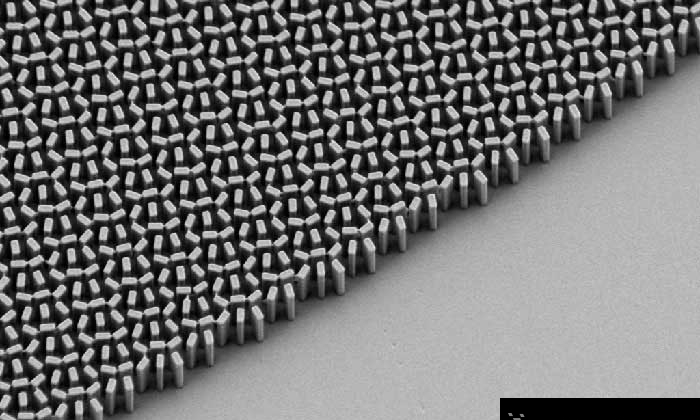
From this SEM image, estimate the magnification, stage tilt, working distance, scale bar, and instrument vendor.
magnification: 22.81 K X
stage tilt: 35°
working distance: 6.7 mm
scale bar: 1000 nm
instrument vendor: Zeiss